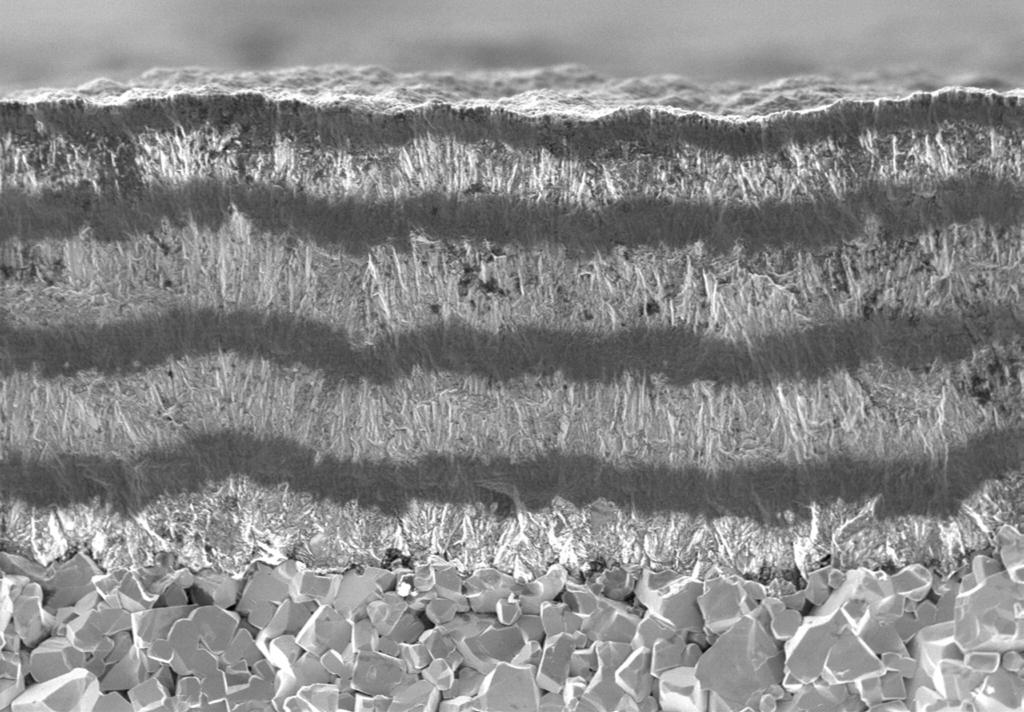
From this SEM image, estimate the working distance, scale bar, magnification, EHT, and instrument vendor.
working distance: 5 mm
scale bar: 5000 nm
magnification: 5 K X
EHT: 2 kV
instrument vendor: Zeiss